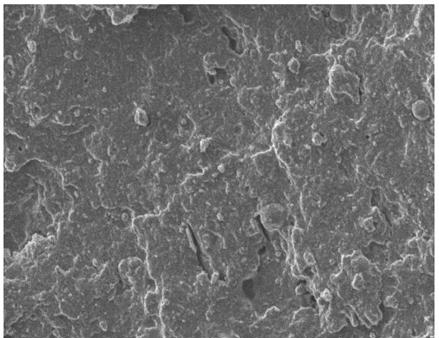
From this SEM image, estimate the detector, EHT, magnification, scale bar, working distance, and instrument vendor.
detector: LEI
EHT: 8 kV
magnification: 0.5 K X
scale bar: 10000 nm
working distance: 8 mm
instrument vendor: JEOL